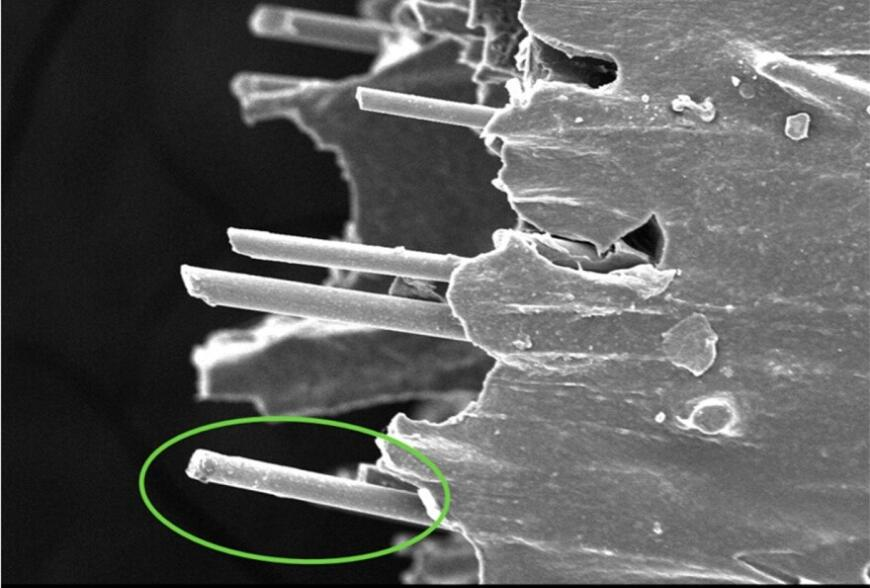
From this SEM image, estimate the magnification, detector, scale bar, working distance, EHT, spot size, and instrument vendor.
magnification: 0.5 K X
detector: SE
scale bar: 100000 nm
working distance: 11.5 mm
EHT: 12 kV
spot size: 9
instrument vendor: other